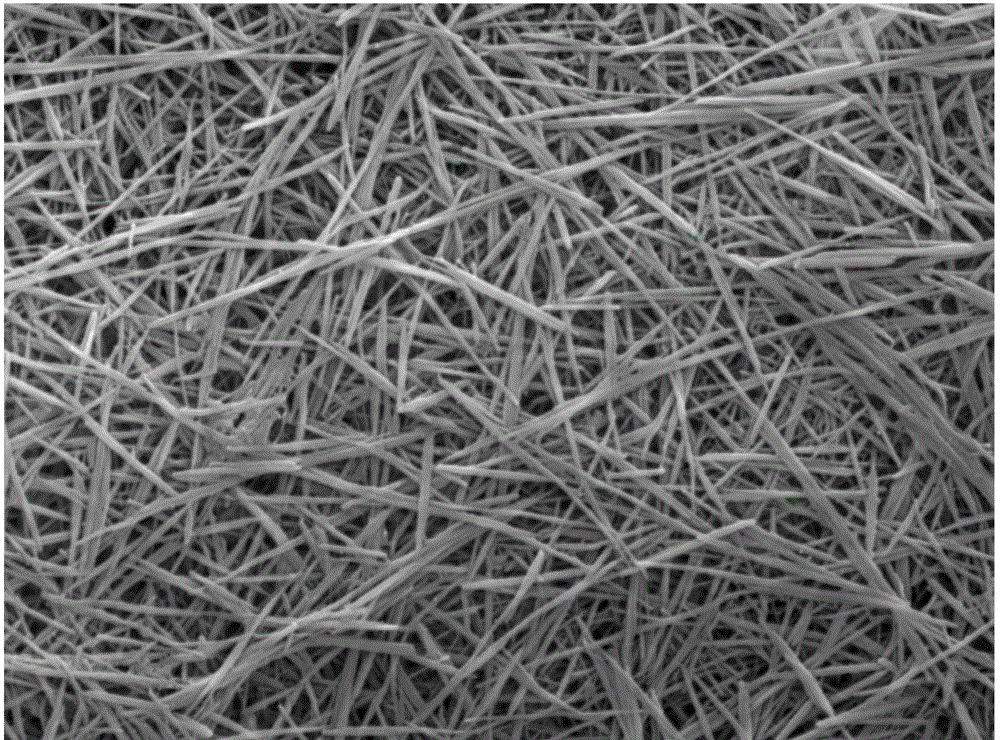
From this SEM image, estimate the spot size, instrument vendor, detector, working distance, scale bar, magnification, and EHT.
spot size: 5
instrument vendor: FEI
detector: SE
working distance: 12.4 mm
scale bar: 2000 nm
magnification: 10 K X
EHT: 20 kV